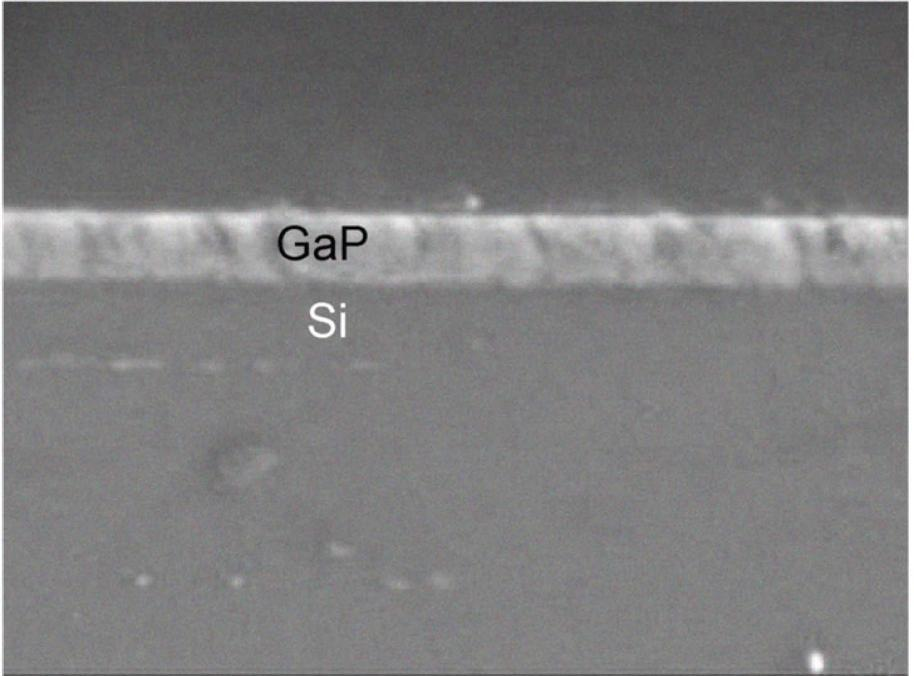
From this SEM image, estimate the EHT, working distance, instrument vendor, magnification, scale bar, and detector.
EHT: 3 kV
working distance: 7.6 mm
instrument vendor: JEOL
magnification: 43 K X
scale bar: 100 nm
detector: LEI